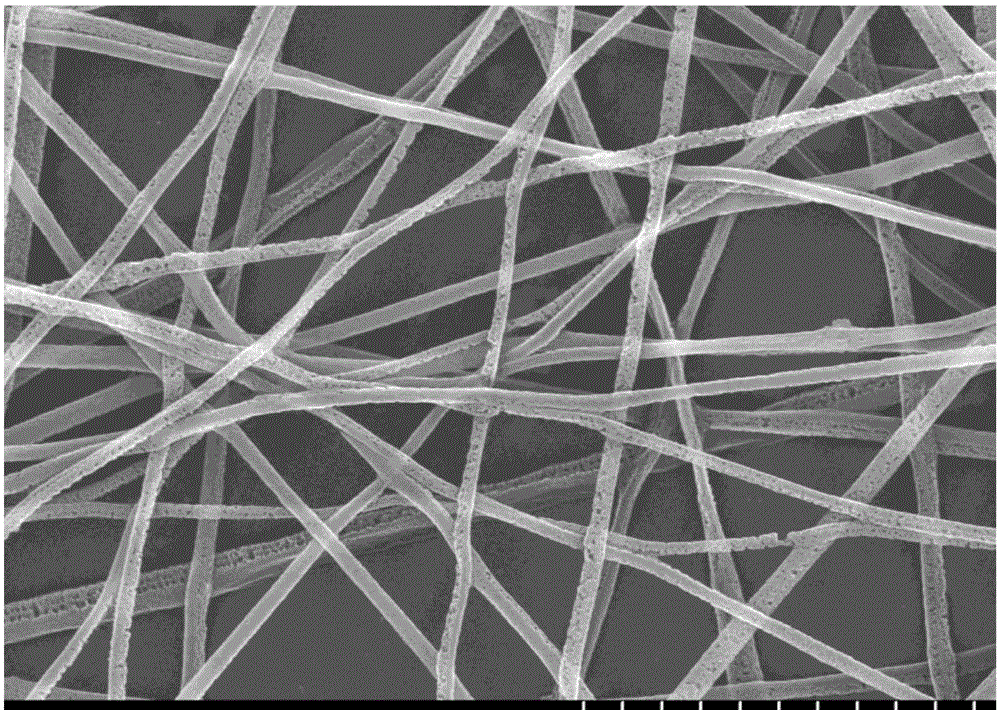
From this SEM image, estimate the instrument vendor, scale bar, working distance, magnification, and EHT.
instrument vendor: Hitachi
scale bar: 10000 nm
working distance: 13.8 mm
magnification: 5 K X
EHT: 20 kV